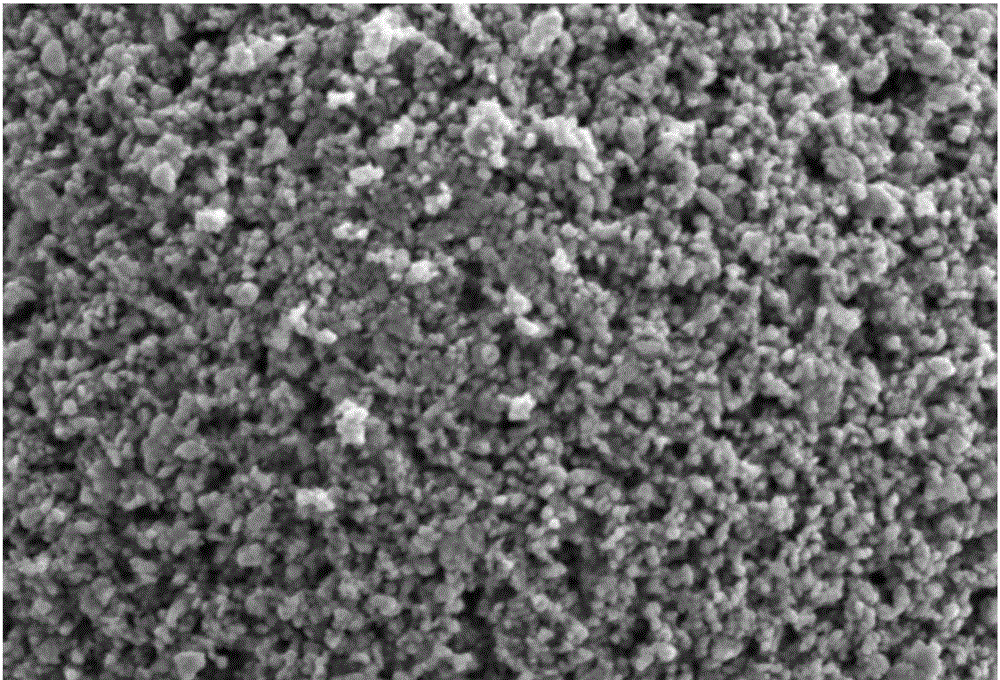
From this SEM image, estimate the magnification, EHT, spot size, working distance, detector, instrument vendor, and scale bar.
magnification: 10 K X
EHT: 5 kV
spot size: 30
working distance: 10 mm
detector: SEI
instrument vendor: JEOL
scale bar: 1000 nm